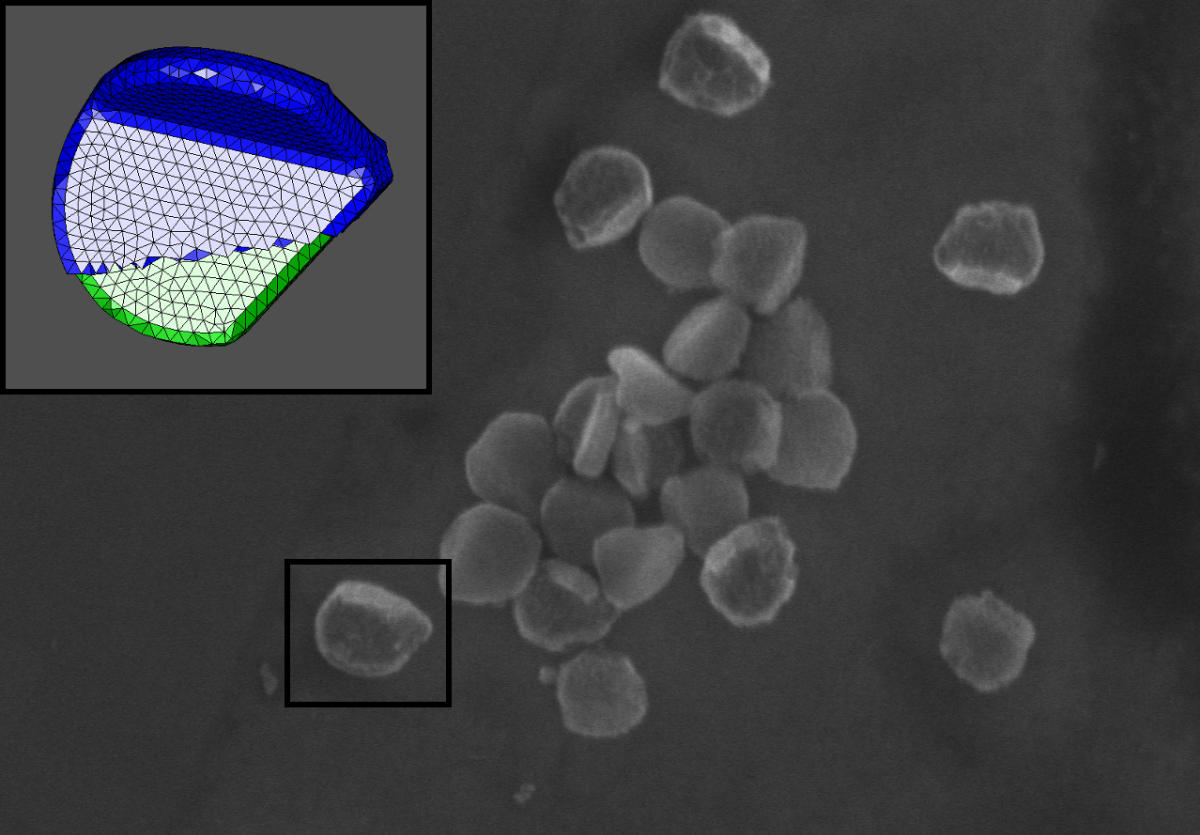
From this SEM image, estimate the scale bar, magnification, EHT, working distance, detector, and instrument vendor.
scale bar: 500 nm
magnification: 70 K X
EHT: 5 kV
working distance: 8.3 mm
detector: SE(U)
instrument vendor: Hitachi